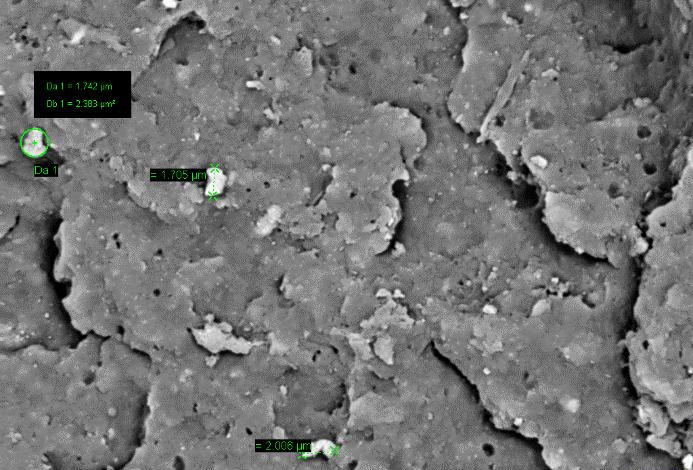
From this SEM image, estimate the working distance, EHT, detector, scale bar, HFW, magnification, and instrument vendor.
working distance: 8 mm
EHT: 10 kV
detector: CZ BSD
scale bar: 2000 nm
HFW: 42.47 µm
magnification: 7 K X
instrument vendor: Zeiss